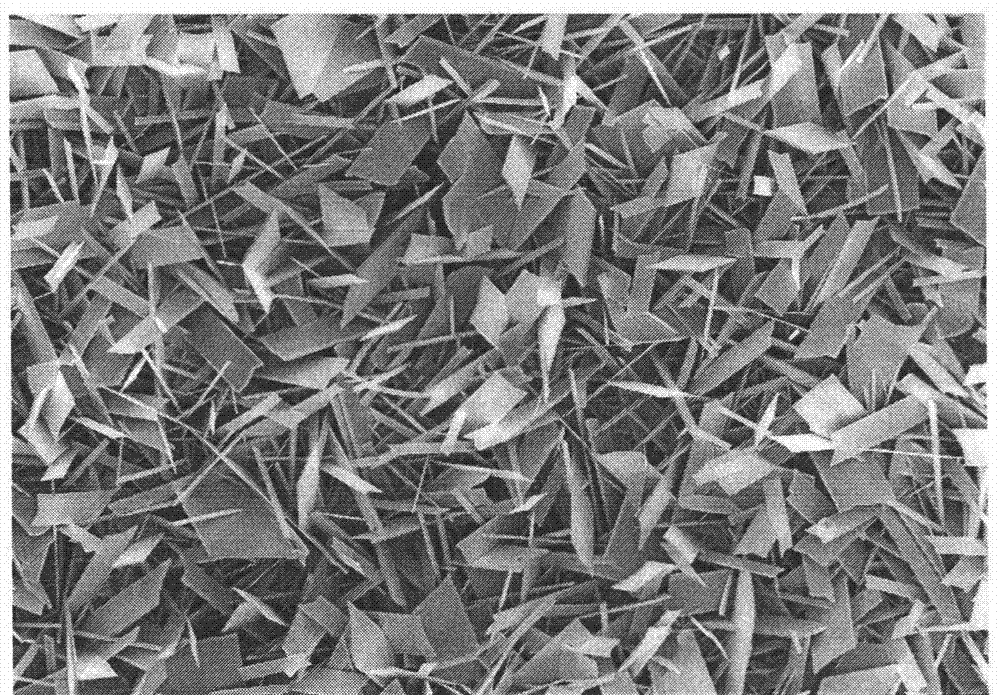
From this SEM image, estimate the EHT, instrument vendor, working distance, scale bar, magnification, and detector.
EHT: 15 kV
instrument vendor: Hitachi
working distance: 10.5 mm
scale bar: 100000 nm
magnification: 0.5 K X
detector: SE(U)